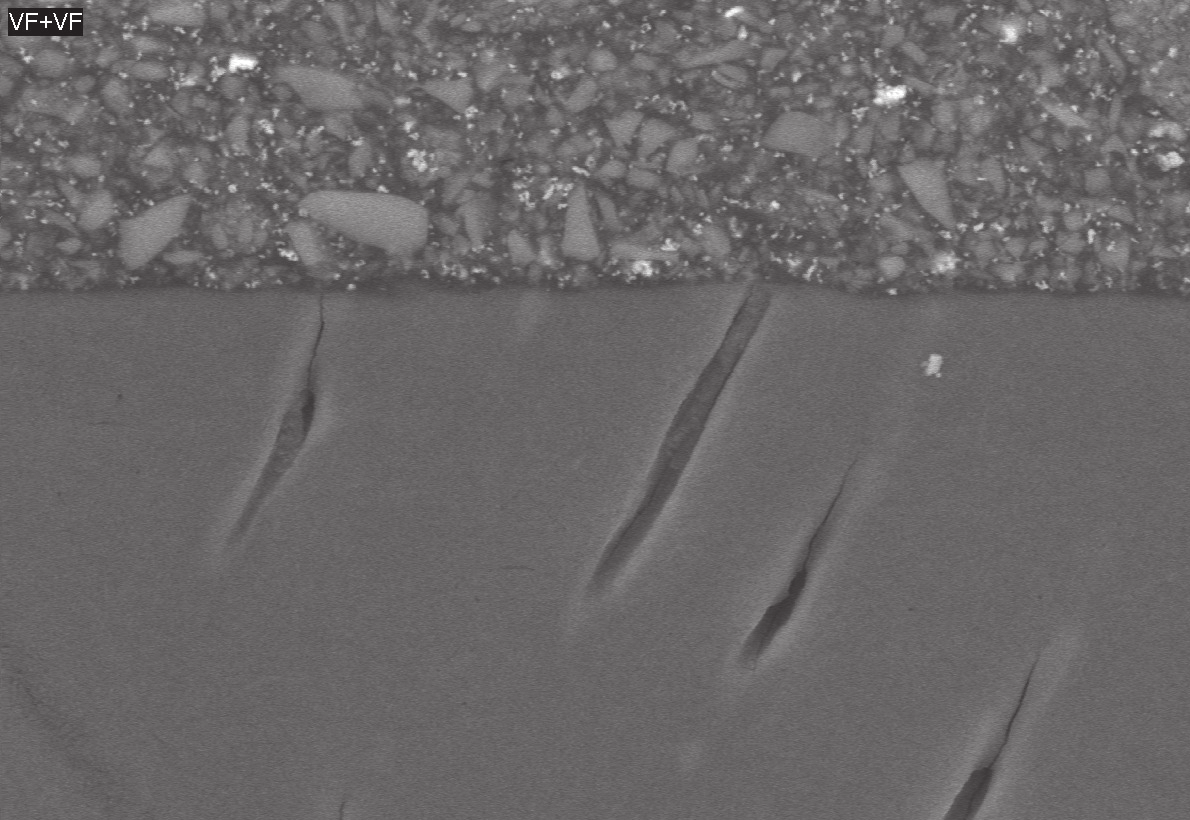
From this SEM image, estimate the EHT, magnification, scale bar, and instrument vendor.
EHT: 20 kV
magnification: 2 K X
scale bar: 15000 nm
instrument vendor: Hitachi